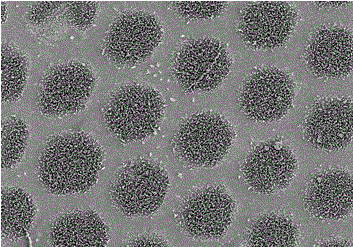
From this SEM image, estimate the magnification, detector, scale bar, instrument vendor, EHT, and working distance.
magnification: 5 K X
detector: SE(M)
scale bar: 10000 nm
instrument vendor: Hitachi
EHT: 3 kV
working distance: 10.1 mm